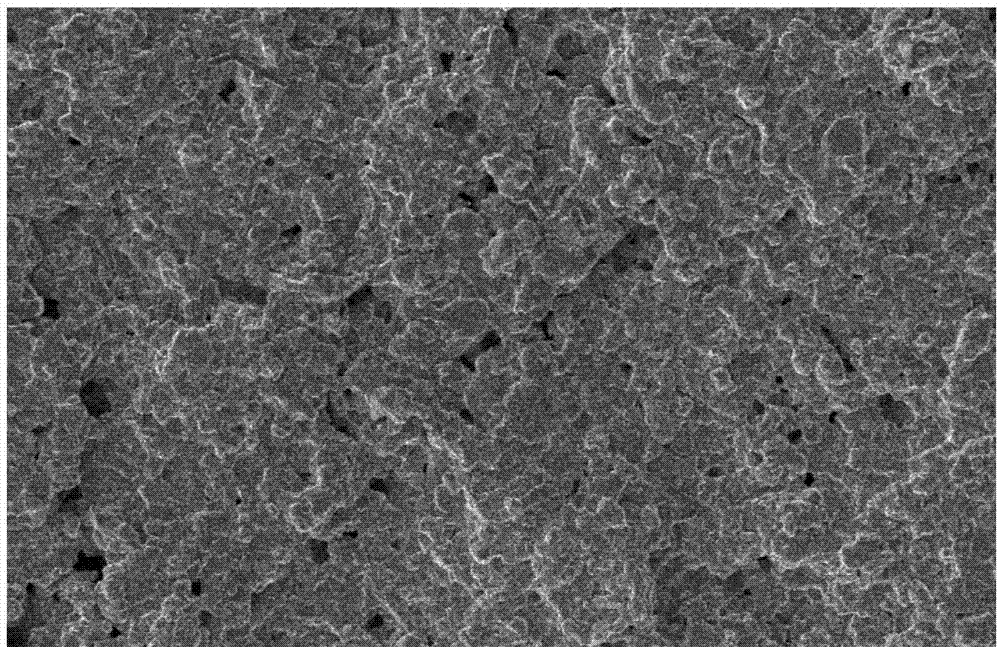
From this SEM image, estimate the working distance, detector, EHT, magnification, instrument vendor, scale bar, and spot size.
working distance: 4.7 mm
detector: SE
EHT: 5 kV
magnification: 4 K X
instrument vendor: FEI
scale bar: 20000 nm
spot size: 3